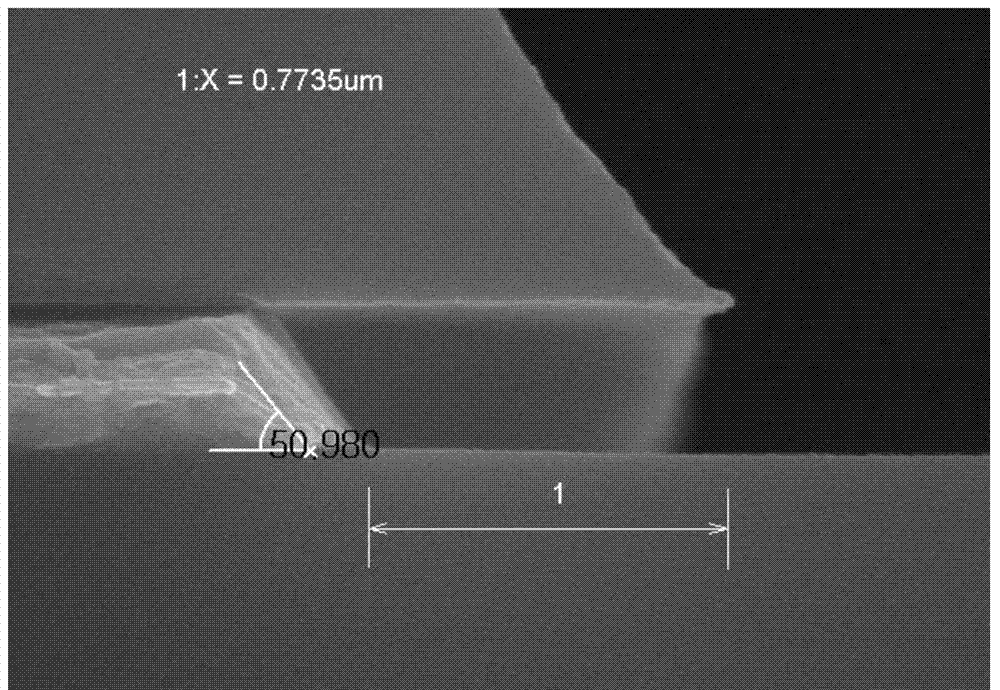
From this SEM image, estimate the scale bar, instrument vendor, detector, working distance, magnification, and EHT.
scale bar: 500 nm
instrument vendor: Hitachi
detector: SE(M)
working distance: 11.1 mm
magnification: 60 K X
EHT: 15 kV